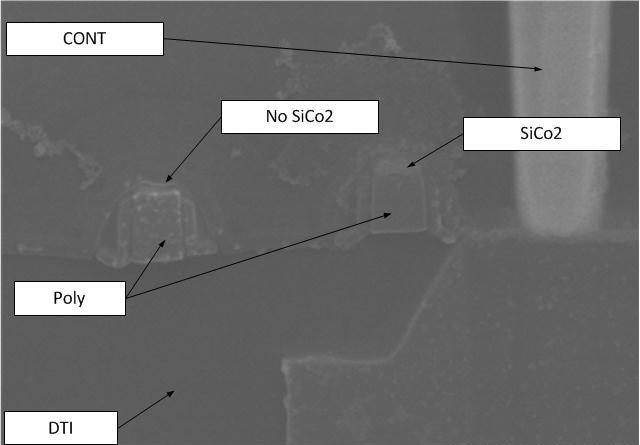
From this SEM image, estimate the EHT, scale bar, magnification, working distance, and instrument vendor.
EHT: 10 kV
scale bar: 500 nm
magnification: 70 K X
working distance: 8.1 mm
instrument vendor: Hitachi